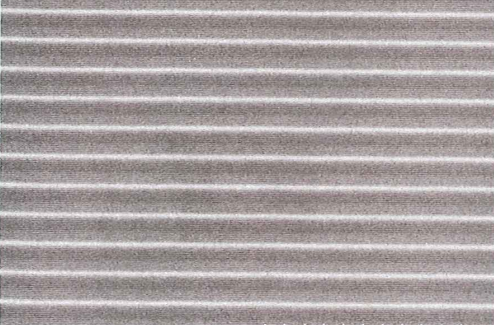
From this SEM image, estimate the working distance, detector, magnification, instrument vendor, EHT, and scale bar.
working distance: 11.5 mm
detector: SE(M)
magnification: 10 K X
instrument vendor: Hitachi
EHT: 0.5 kV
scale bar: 5000 nm